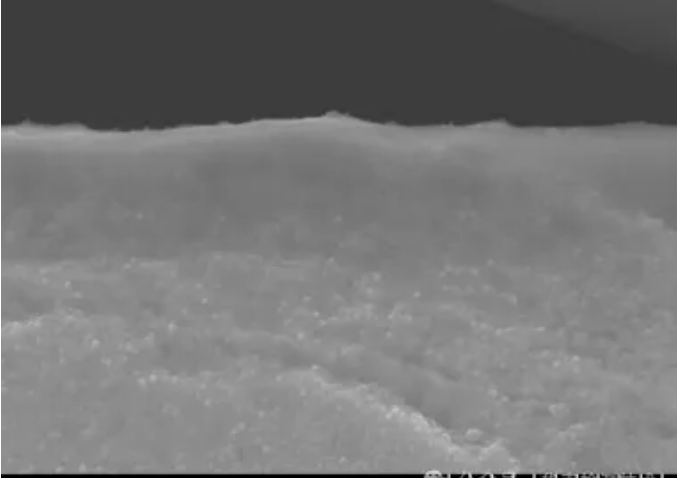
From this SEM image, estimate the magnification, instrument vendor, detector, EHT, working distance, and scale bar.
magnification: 20 K X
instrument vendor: Hitachi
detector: SE(U)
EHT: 15 kV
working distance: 14.7 mm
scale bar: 2000 nm